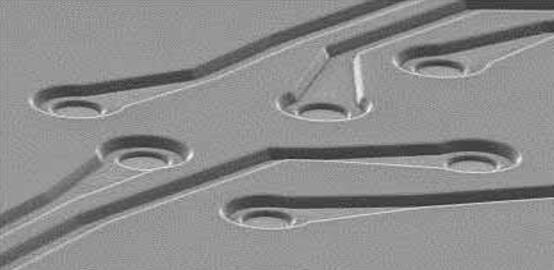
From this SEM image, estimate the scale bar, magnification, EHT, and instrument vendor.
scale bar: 50000 nm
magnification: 0.35 K X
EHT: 15 kV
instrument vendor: JEOL